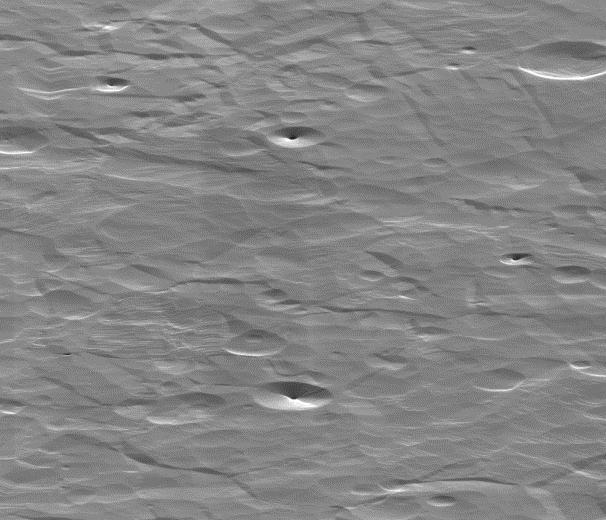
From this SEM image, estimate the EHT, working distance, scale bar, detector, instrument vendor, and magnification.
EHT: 30 kV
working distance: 13.1 mm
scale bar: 5000 nm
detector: ETD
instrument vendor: FEI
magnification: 7.5 K X